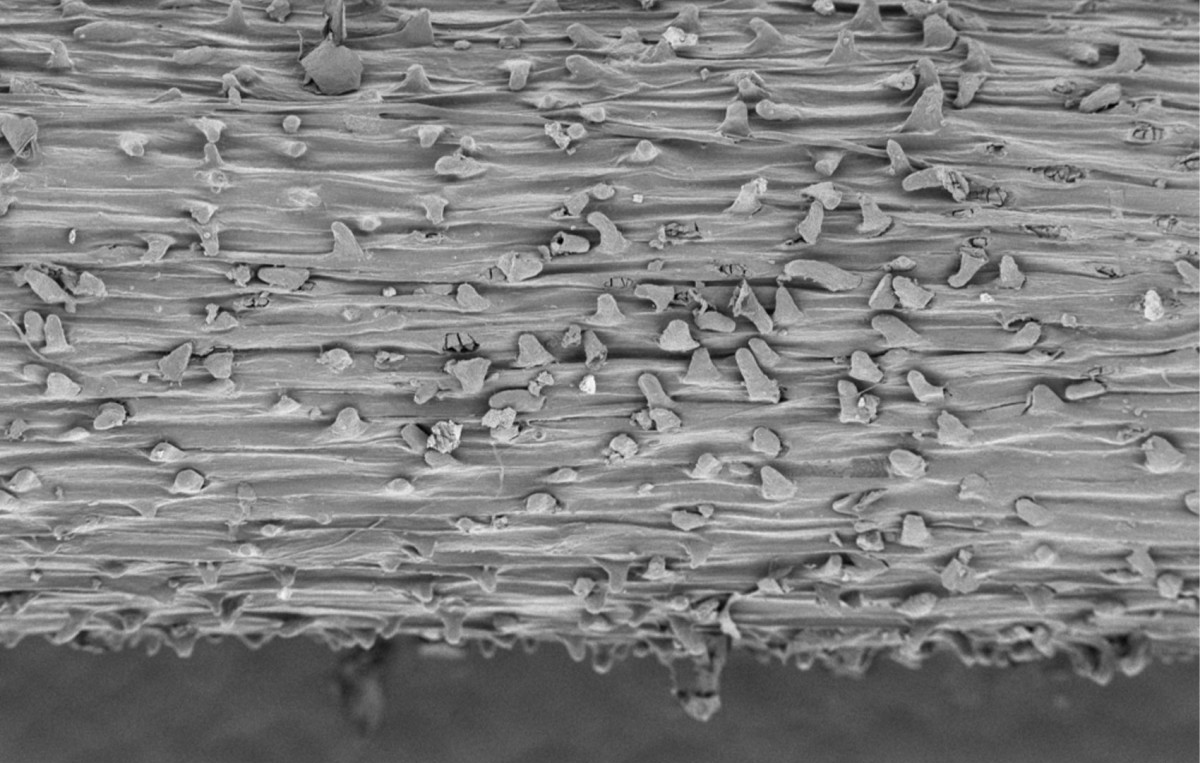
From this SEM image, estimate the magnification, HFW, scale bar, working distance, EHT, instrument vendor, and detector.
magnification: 0.35 K X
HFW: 789 µm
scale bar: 200000 nm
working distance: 5 mm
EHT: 1 kV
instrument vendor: Thermo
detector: T1